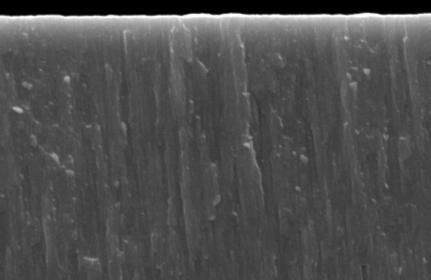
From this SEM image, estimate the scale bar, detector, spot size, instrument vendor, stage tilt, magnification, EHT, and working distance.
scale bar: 1000 nm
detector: SE1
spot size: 350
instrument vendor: Zeiss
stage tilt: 0°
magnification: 100 K X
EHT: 20 kV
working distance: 19 mm